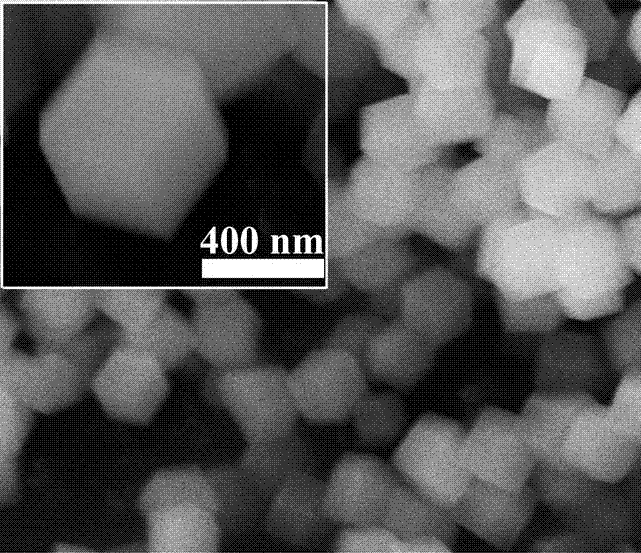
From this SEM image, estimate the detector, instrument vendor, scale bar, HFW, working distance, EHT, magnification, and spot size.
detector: ETD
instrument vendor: FEI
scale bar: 1000 nm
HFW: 6.22 µm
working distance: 9.8 mm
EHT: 10 kV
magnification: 24 K X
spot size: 2